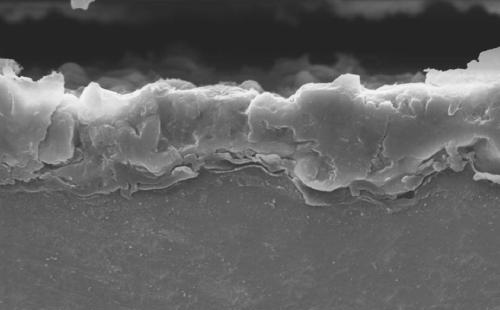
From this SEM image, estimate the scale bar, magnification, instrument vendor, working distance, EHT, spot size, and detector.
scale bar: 5000 nm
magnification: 2 K X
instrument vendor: FEI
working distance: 14 mm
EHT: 20 kV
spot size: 4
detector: SE